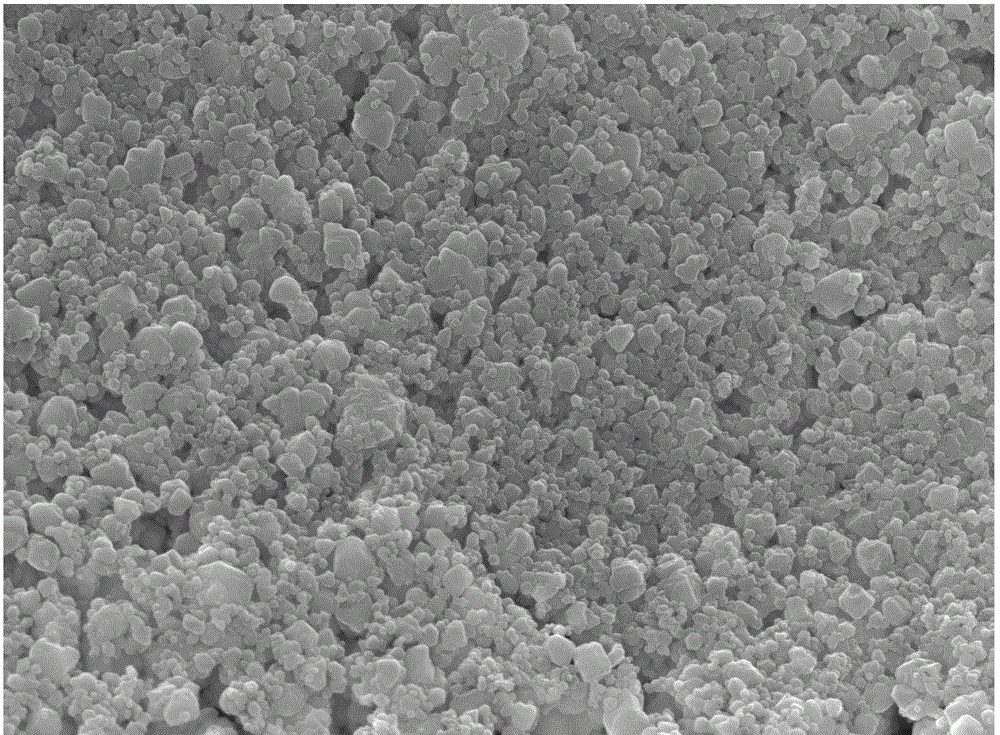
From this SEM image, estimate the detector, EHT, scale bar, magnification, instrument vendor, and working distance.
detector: SEI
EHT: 15 kV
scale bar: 1000 nm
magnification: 10 K X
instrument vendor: JEOL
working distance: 9.5 mm